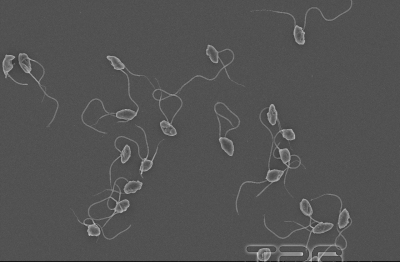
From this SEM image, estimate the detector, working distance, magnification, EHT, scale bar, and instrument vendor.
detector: SE(U)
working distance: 7.5 mm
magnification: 2 K X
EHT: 5 kV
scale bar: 20000 nm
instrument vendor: Hitachi